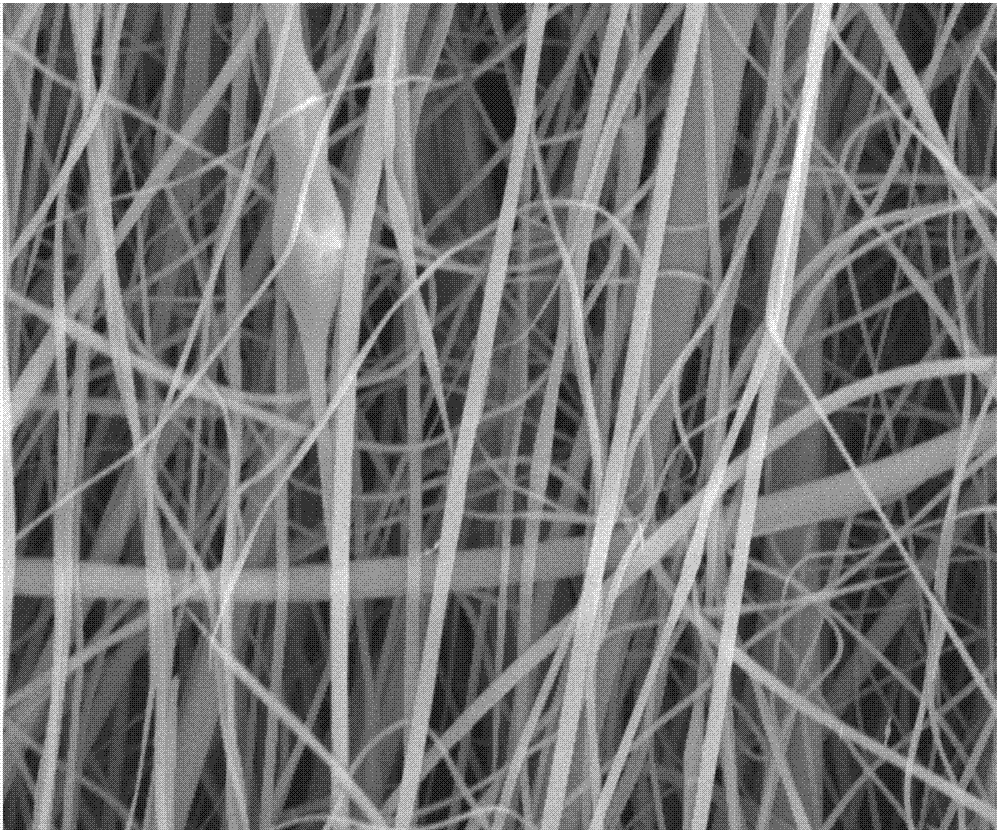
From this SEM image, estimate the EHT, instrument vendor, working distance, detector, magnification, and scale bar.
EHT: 10 kV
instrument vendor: FEI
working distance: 5.2 mm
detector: ETD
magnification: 10 K X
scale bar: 5000 nm